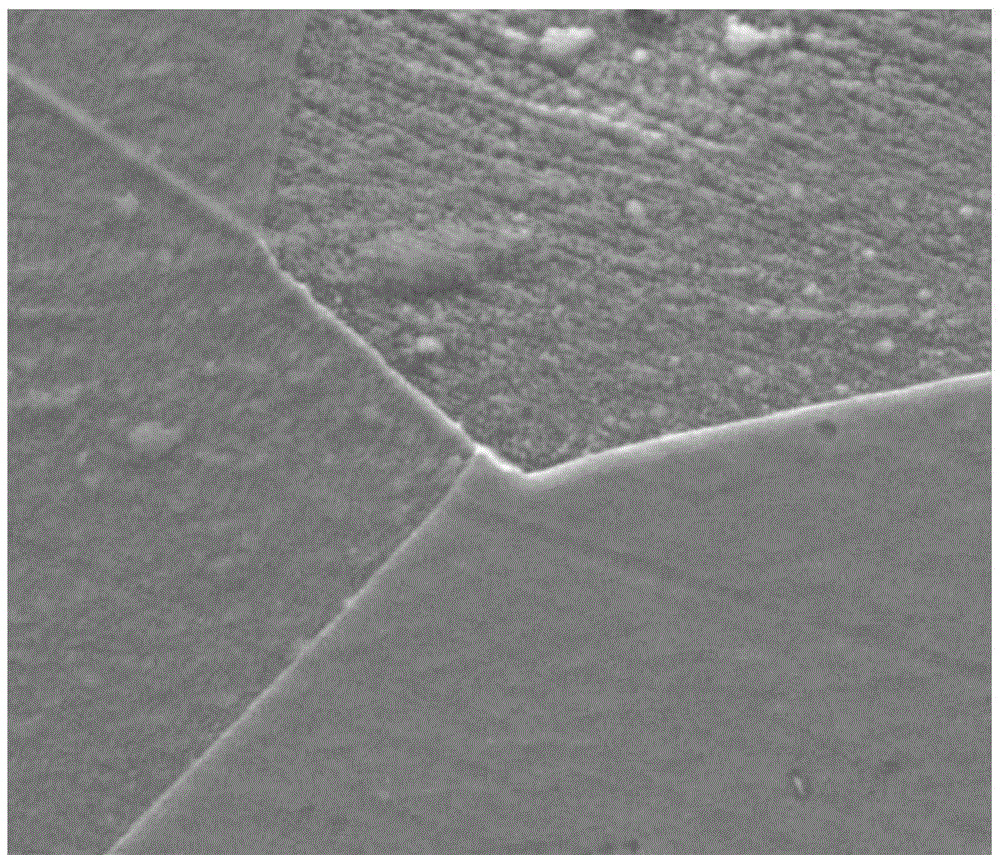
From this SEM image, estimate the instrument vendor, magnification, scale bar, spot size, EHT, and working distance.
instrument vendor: FEI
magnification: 5 K X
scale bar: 10000 nm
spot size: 4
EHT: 20 kV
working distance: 11.1 mm